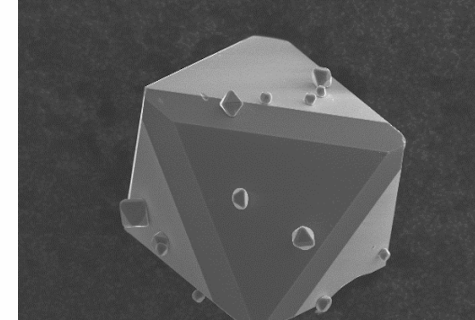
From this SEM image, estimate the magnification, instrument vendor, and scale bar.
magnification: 0.6 K X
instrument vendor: JEOL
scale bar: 30000 nm